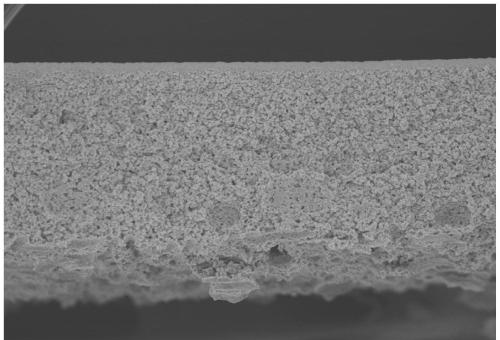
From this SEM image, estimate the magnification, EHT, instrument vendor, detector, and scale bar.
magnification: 0.3 K X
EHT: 4 kV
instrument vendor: Hitachi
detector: SE(U)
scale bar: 100000 nm